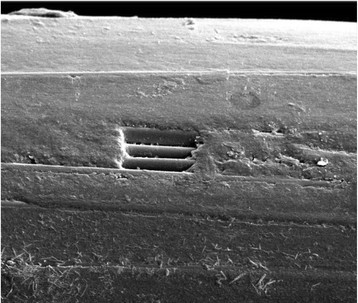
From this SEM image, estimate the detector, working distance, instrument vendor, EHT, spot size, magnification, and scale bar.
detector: ETD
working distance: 10.9 mm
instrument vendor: FEI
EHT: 10 kV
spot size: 3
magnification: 1.6 K X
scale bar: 100000 nm